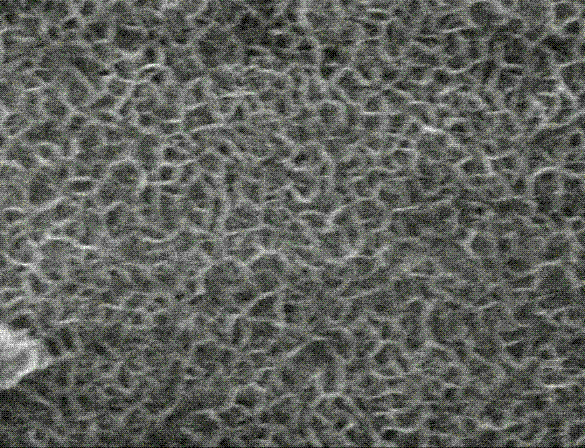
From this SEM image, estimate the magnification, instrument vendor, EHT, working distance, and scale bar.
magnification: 20 K X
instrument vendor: JEOL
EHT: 15 kV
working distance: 13.9 mm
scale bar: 1000 nm